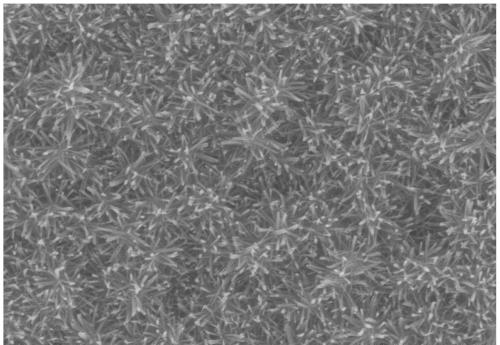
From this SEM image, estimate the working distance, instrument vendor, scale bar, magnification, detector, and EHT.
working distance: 11.5 mm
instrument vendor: Hitachi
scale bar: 1000 nm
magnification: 30 K X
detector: SE(M)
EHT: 10 kV